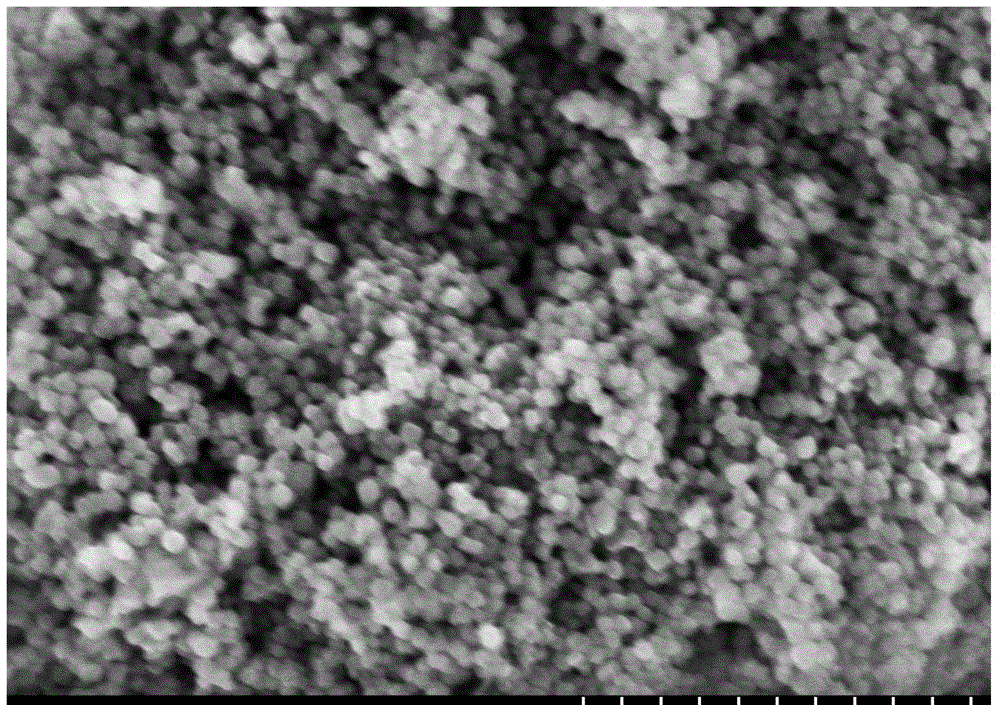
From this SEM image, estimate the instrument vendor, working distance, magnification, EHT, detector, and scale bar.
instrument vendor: Hitachi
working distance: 4.1 mm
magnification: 100 K X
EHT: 5 kV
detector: SE(U,LA10)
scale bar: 500 nm